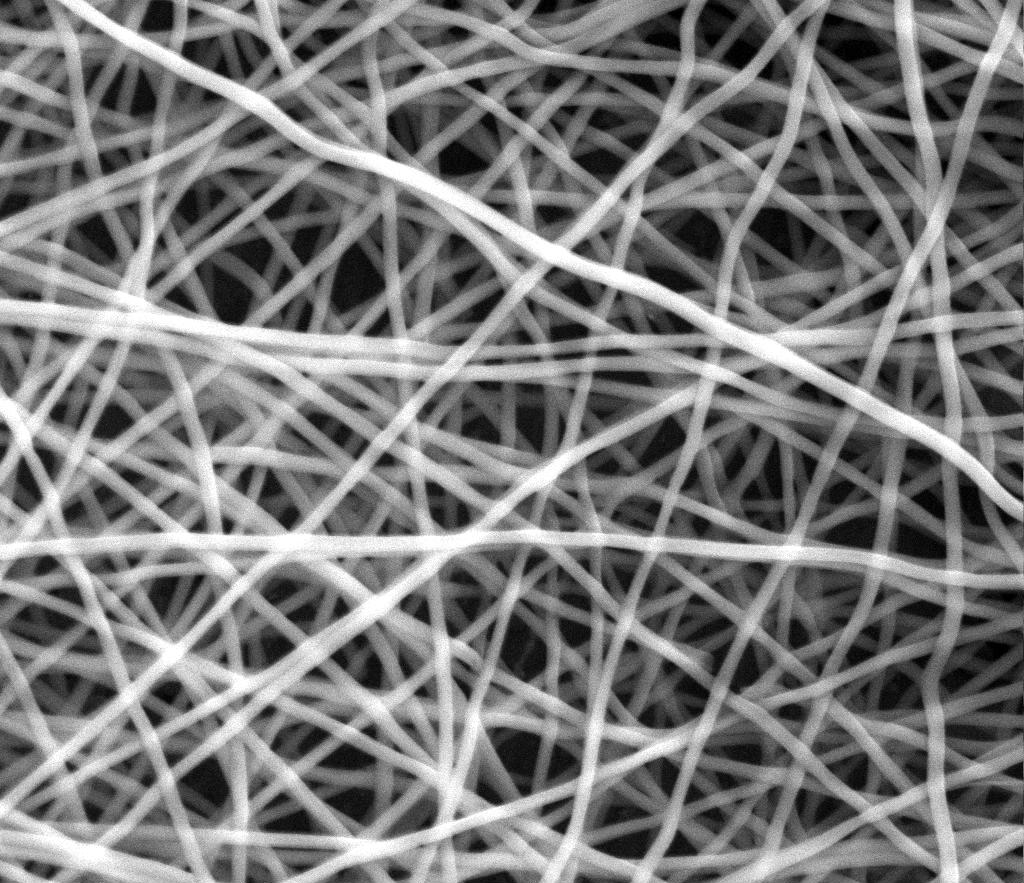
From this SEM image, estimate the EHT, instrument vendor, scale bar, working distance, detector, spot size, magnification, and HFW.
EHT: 15 kV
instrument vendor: FEI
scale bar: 20000 nm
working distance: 16.6 mm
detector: ETD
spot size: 3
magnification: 5 K X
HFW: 54.08 µm